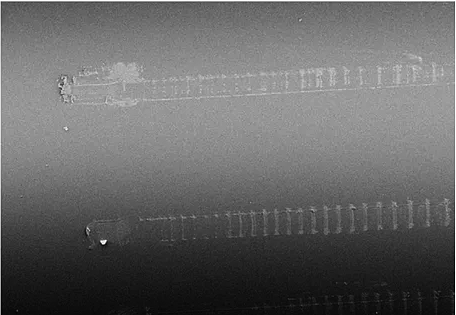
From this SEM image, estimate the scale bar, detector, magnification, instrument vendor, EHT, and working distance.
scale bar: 100000 nm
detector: SE1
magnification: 0.043 K X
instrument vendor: Zeiss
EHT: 8 kV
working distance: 19 mm